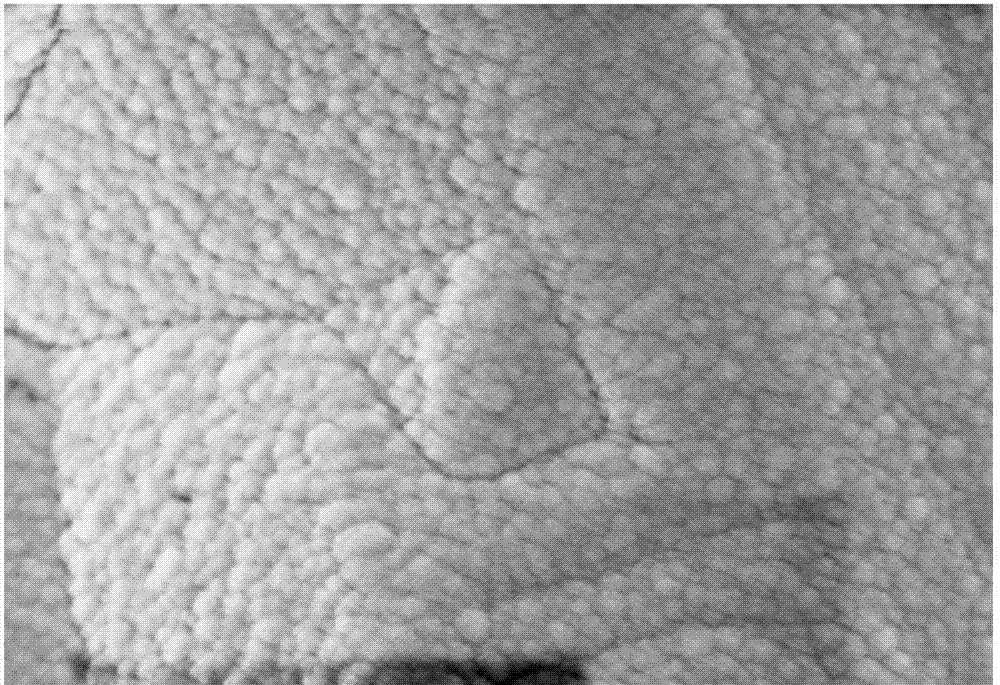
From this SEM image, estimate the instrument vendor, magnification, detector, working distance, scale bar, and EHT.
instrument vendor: Zeiss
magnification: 20 K X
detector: InLens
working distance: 5.7 mm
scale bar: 200 nm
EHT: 5 kV